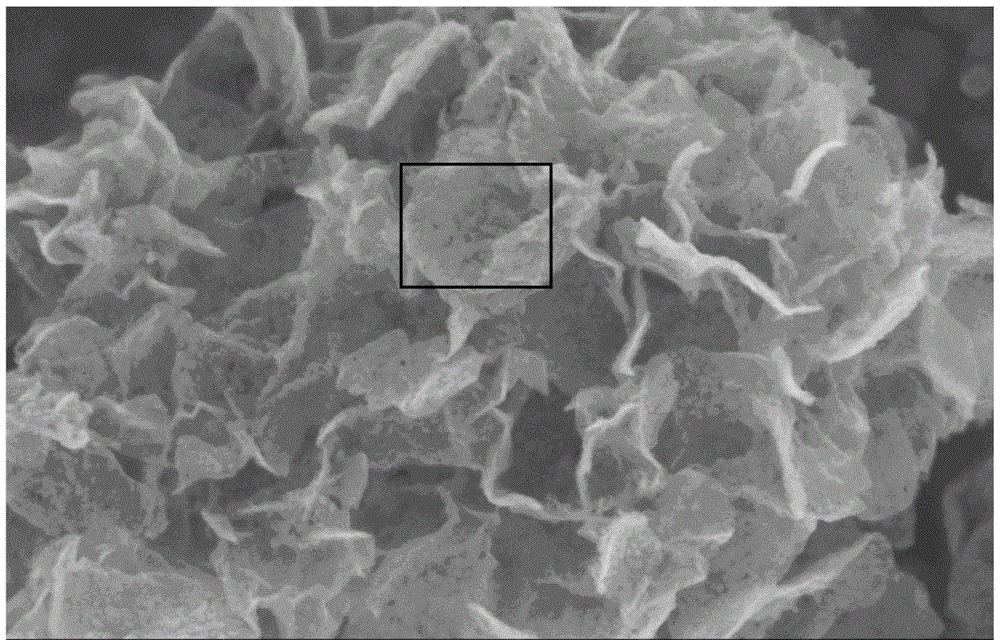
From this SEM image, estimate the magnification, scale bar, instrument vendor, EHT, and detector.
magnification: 60 K X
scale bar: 500 nm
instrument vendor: Hitachi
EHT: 4 kV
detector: SE(U)